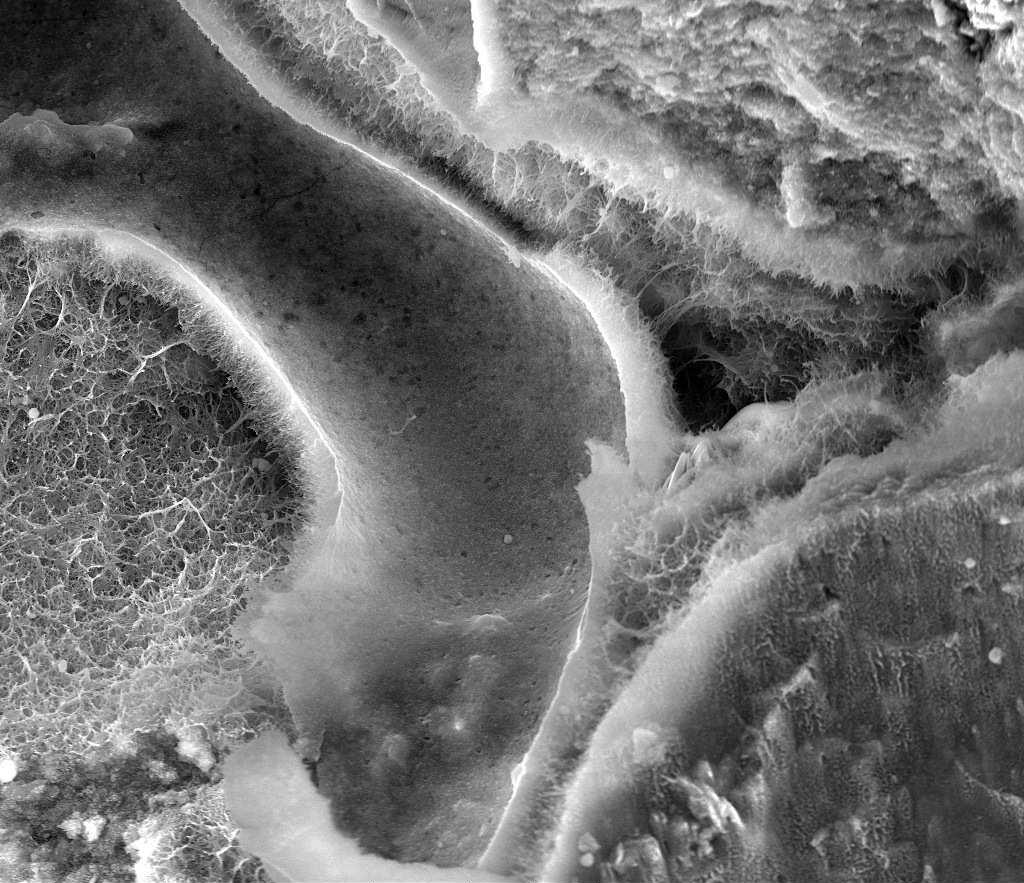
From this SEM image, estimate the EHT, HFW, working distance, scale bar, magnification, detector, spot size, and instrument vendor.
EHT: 18 kV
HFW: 149 µm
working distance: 6.4 mm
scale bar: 50000 nm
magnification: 2 K X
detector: LVD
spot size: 4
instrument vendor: FEI